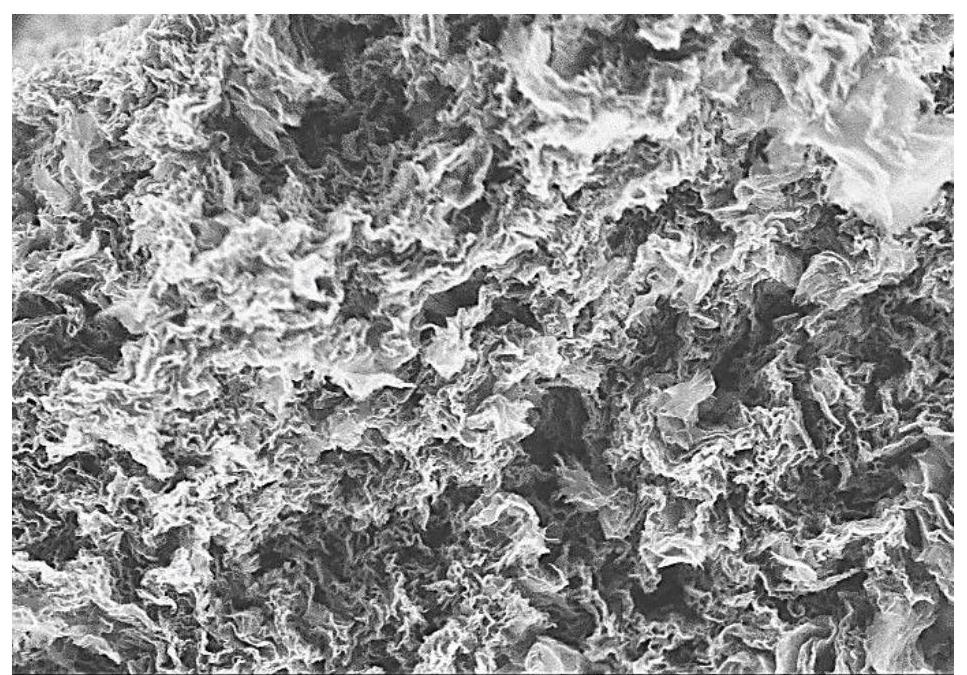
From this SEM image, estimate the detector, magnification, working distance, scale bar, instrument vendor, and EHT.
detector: SE(M)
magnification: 10 K X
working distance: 9.3 mm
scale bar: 5000 nm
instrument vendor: Hitachi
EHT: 5 kV